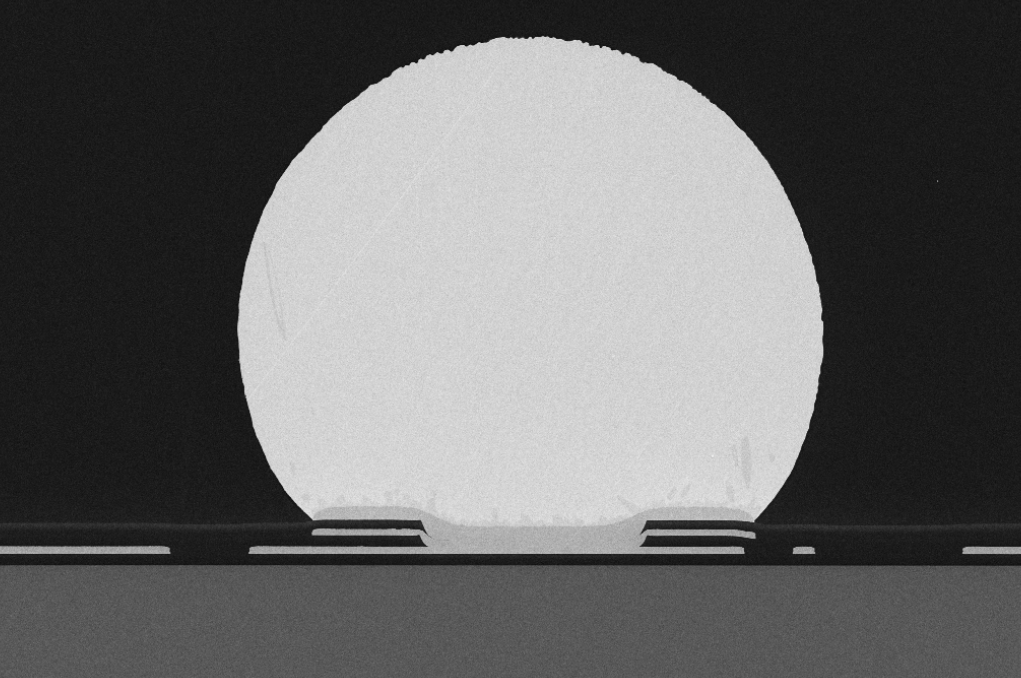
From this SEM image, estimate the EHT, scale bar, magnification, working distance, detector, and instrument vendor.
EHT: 10 kV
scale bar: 20000 nm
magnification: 0.25 K X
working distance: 9.3 mm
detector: SE2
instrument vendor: Zeiss